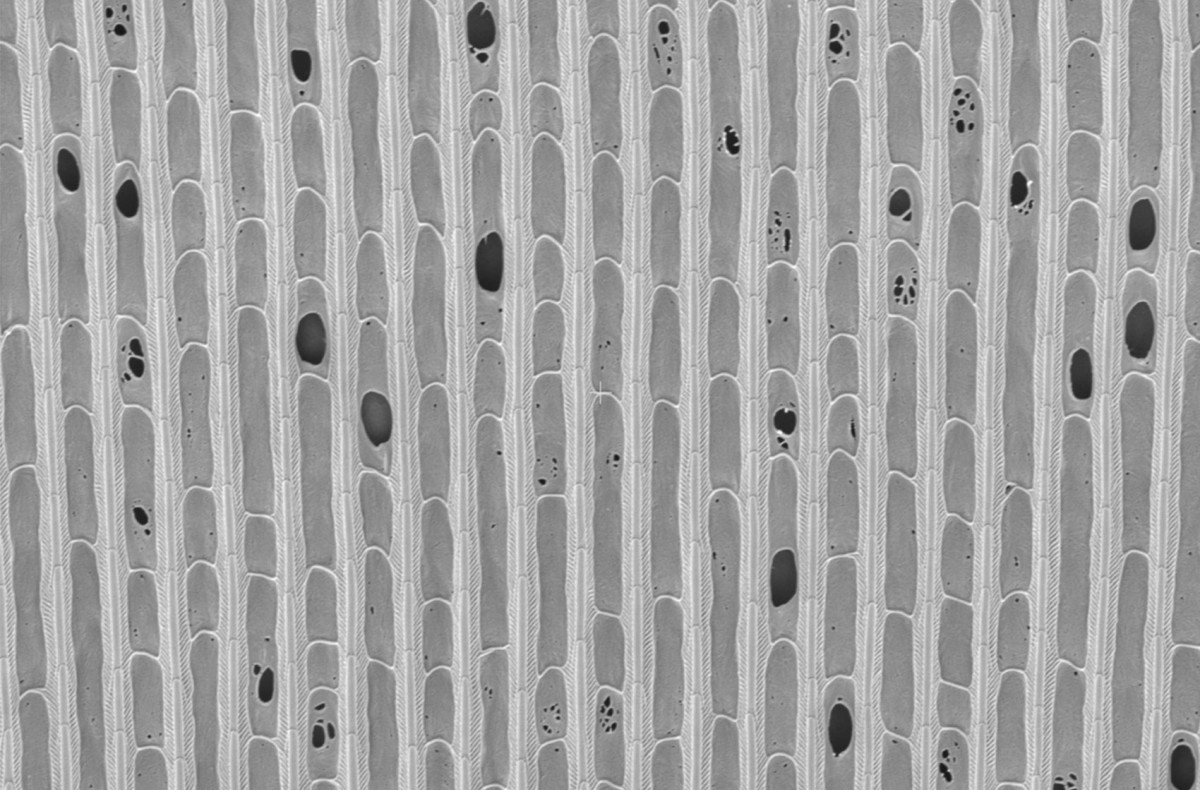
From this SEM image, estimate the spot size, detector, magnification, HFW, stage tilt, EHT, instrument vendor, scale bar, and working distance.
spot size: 10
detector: ETD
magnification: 10 K X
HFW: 41.4 µm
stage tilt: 0°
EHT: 5 kV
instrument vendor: FEI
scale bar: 10000 nm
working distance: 7.1 mm